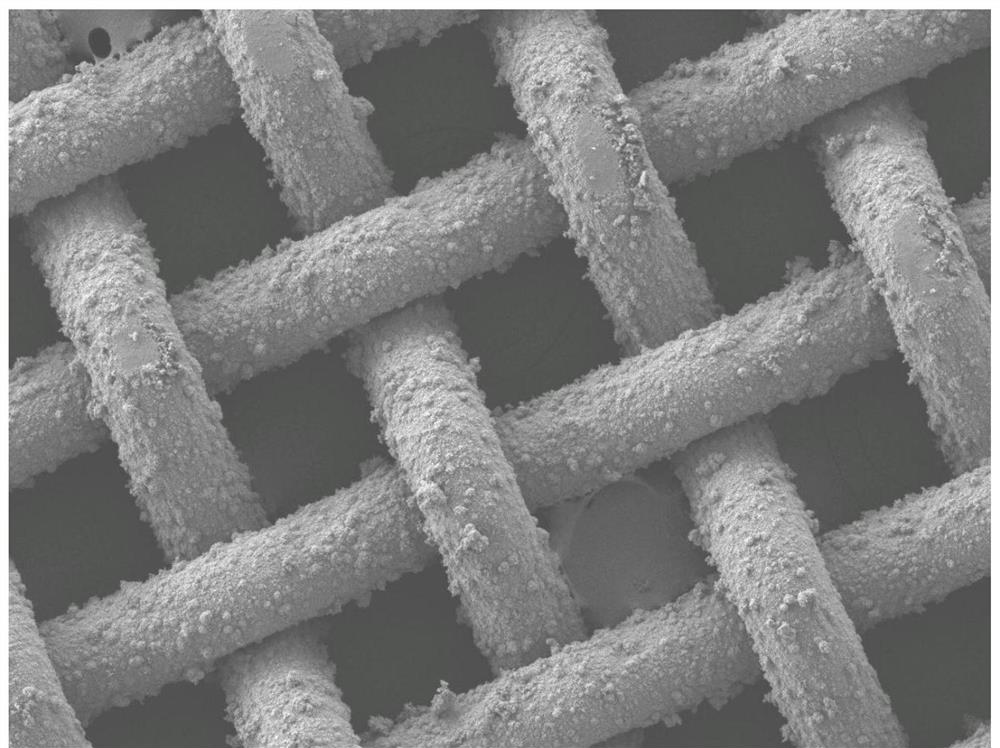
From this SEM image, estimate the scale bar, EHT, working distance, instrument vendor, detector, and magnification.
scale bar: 100000 nm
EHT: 5 kV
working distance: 8.6 mm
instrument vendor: JEOL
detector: LEI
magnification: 0.15 K X